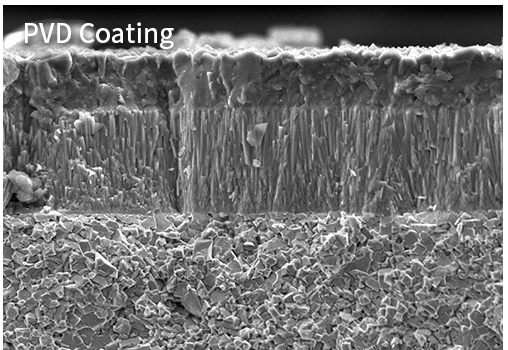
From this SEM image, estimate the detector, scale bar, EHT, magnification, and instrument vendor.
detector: SE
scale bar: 10000 nm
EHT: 10 kV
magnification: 3.5 K X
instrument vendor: other